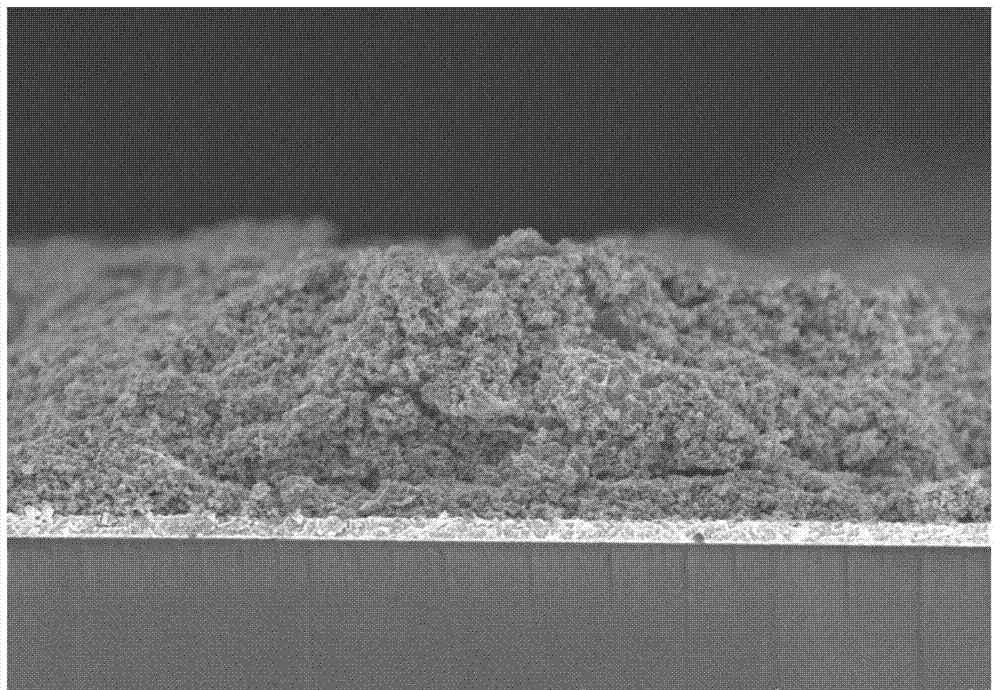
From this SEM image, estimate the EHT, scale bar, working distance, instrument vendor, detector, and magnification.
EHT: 5 kV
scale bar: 10000 nm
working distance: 9.2 mm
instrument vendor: Hitachi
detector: SE(U)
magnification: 5 K X